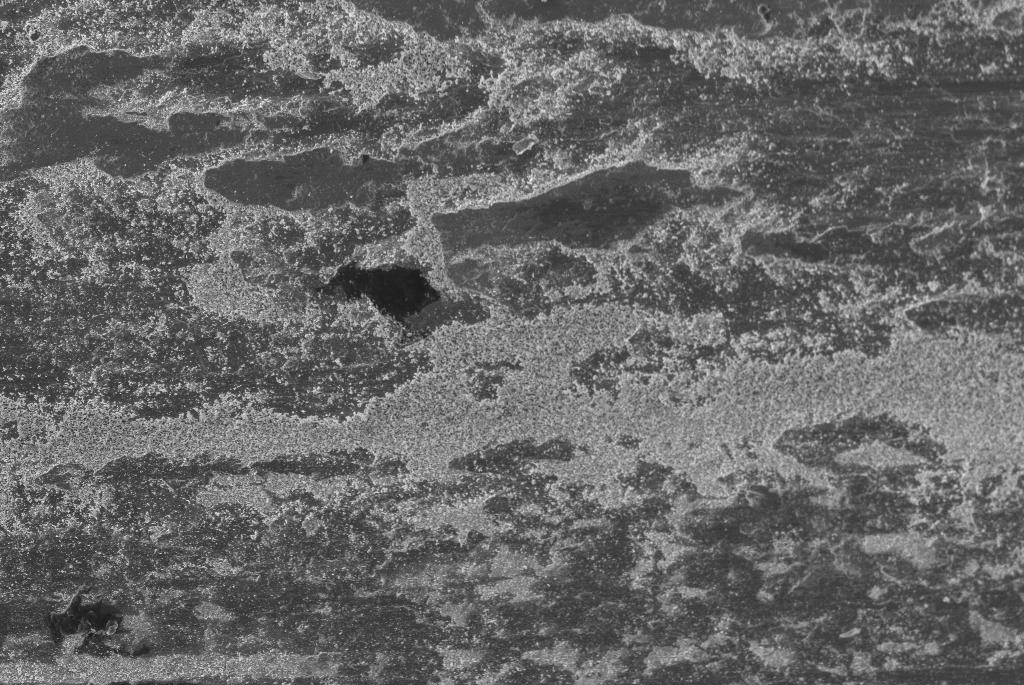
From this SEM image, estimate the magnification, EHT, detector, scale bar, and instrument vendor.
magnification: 0.1 K X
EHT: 20 kV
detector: SE1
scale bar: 200000 nm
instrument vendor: Zeiss